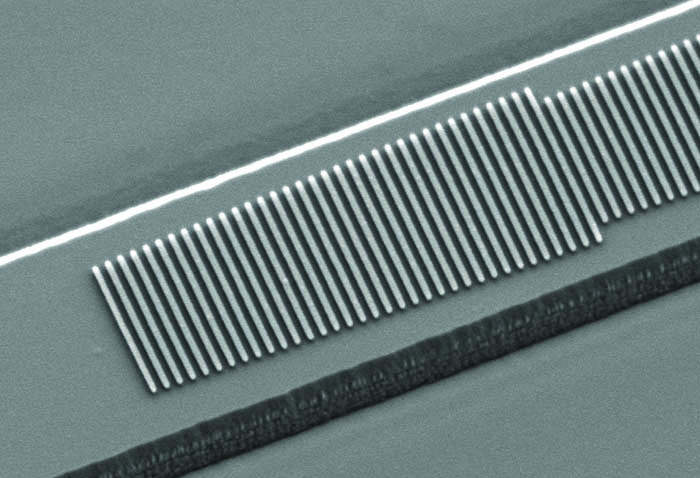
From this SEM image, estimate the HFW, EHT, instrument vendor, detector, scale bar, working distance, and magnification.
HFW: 7.057 µm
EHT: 2.5 kV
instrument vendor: Zeiss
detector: SE2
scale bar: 200 nm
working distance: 6.9 mm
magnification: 42.6 K X